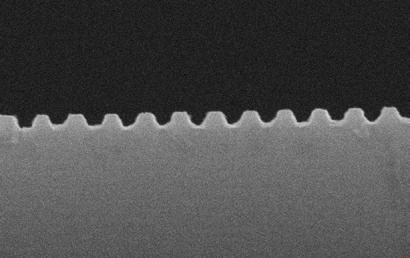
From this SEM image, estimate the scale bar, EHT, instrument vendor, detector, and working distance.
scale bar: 200 nm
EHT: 20 kV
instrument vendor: Zeiss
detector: SE1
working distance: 5 mm